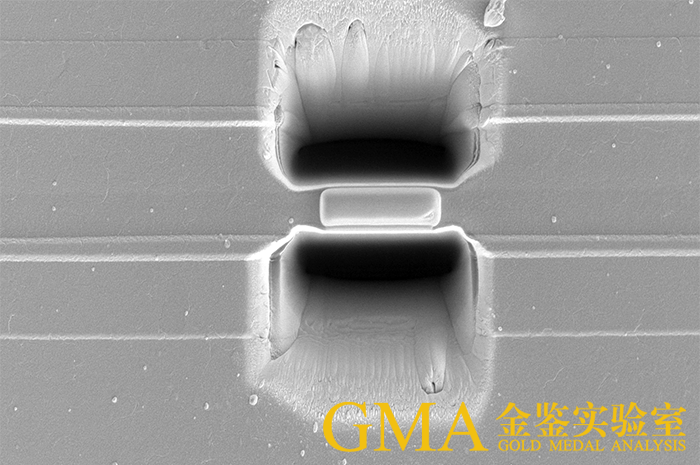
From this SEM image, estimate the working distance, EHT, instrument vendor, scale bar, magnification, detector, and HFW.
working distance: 7 mm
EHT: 5 kV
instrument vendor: FEI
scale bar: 20000 nm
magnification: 2.5 K X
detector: ETD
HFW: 50.8 µm